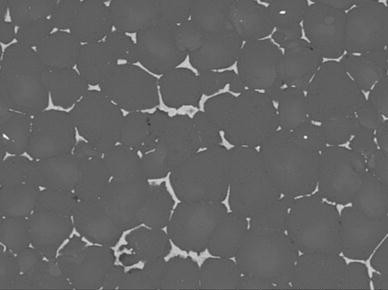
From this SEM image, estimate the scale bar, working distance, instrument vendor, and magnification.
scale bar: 200000 nm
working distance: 8.3 mm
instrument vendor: Hitachi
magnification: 0.5 K X